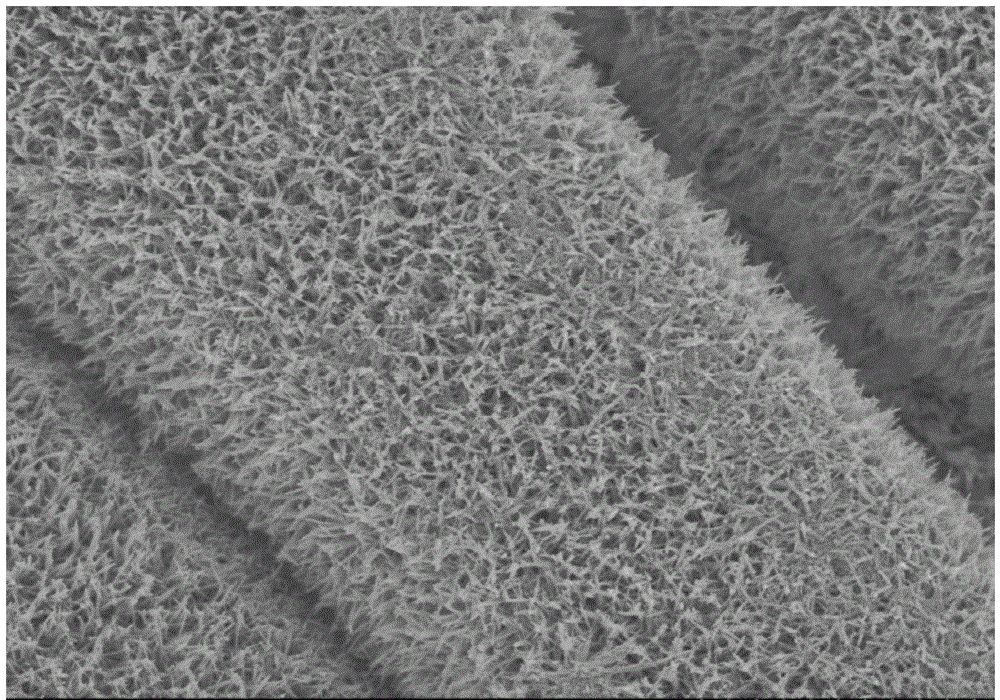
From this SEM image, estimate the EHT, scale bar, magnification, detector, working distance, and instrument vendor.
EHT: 5 kV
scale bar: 5000 nm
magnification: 7 K X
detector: SE(M)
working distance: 6.8 mm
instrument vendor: Hitachi